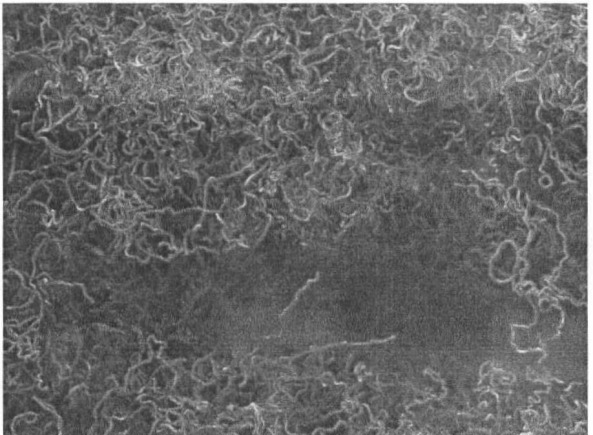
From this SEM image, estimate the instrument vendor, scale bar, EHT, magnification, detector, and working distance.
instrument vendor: JEOL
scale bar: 1000 nm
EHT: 10 kV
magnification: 20 K X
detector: SEI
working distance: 8 mm